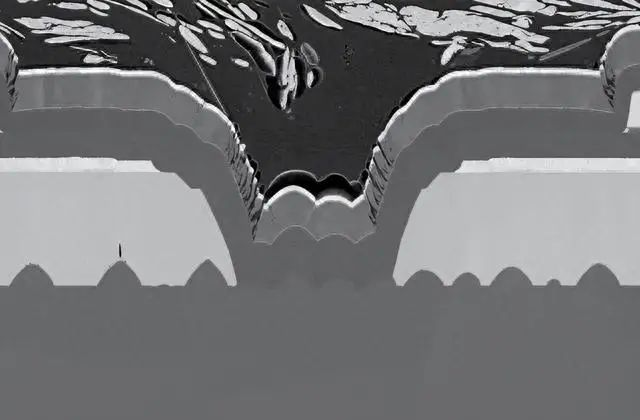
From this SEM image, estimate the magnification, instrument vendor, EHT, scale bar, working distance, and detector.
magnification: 3 K X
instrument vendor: Zeiss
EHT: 2 kV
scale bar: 2000 nm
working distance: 4.9 mm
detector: SE2